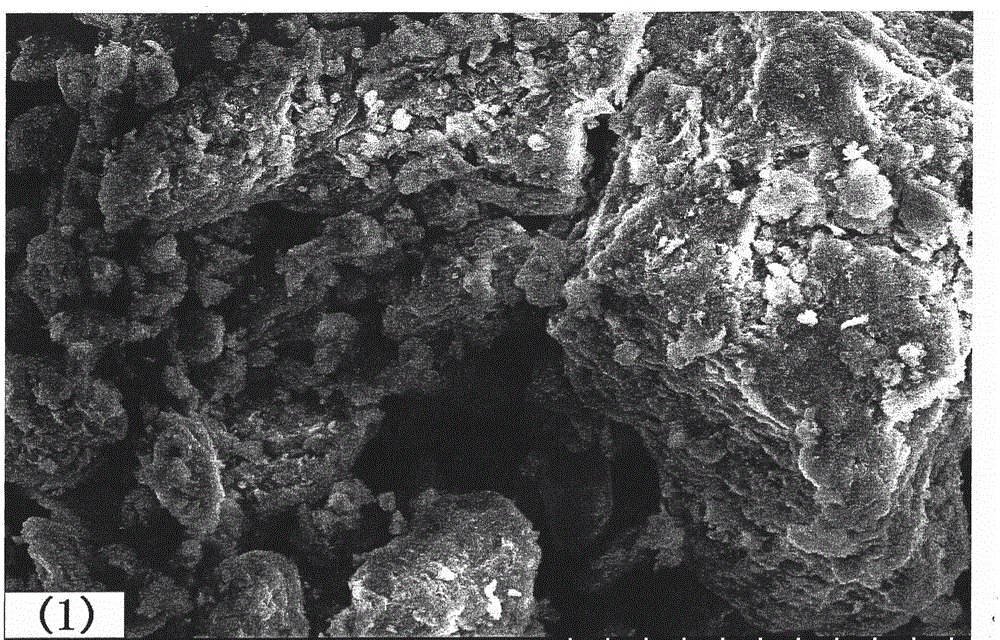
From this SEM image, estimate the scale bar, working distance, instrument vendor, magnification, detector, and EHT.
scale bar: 50000 nm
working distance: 9.8 mm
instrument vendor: Hitachi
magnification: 1 K X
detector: SE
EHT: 15 kV